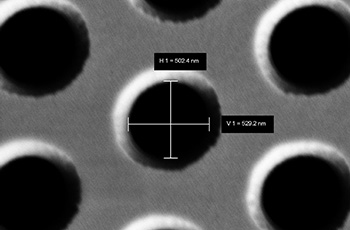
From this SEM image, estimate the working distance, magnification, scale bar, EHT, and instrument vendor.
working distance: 5.1 mm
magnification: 50 K X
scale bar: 200 nm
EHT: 2 kV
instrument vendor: Zeiss